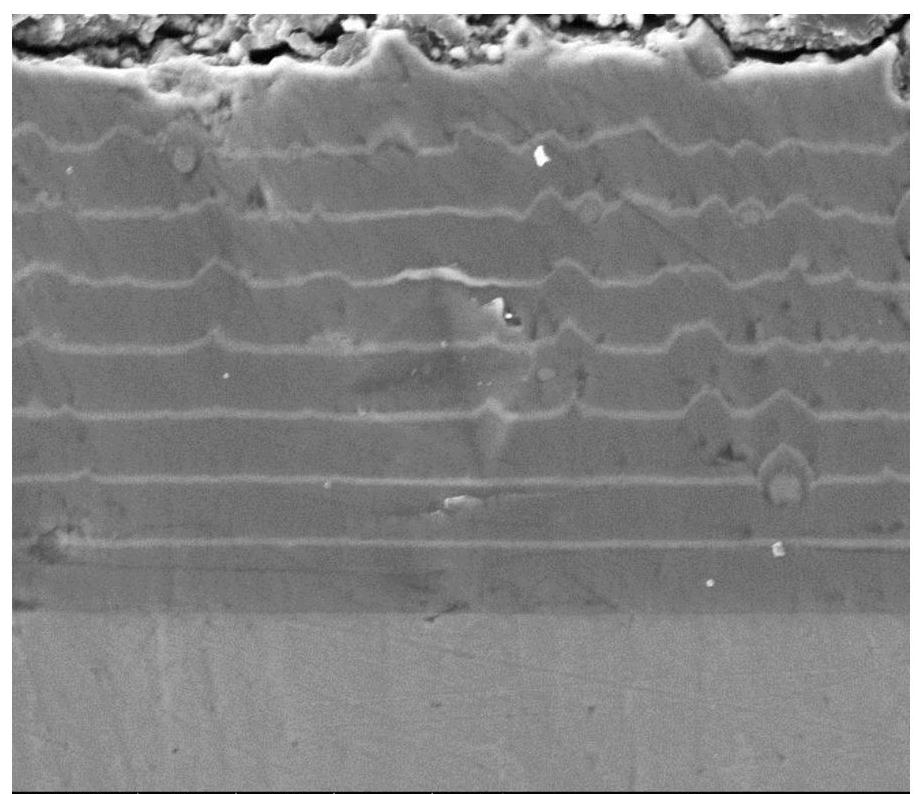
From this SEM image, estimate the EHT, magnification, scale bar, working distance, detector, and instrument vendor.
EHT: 10 kV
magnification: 10 K X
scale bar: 10000 nm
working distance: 10 mm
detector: SE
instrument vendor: FEI